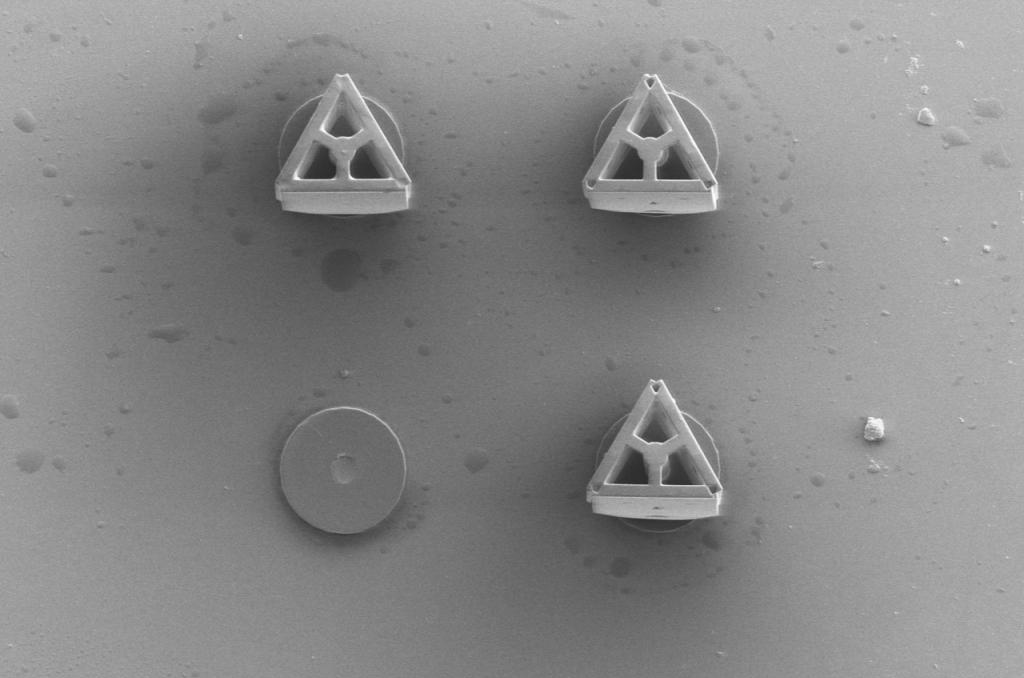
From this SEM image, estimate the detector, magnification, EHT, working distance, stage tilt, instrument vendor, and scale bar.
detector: ETD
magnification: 0.5 K X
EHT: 5 kV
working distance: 8.6 mm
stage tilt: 0°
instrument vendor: FEI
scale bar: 200000 nm